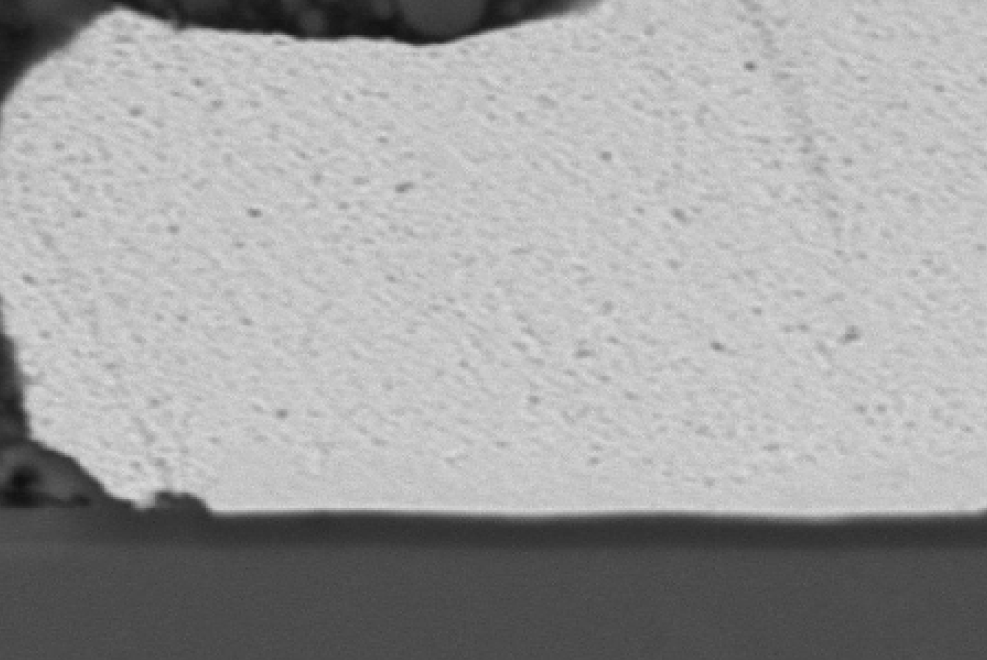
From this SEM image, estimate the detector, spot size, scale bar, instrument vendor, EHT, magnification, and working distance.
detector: SEI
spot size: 48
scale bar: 1000 nm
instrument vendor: JEOL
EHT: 15 kV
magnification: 3.7 K X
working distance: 35 mm